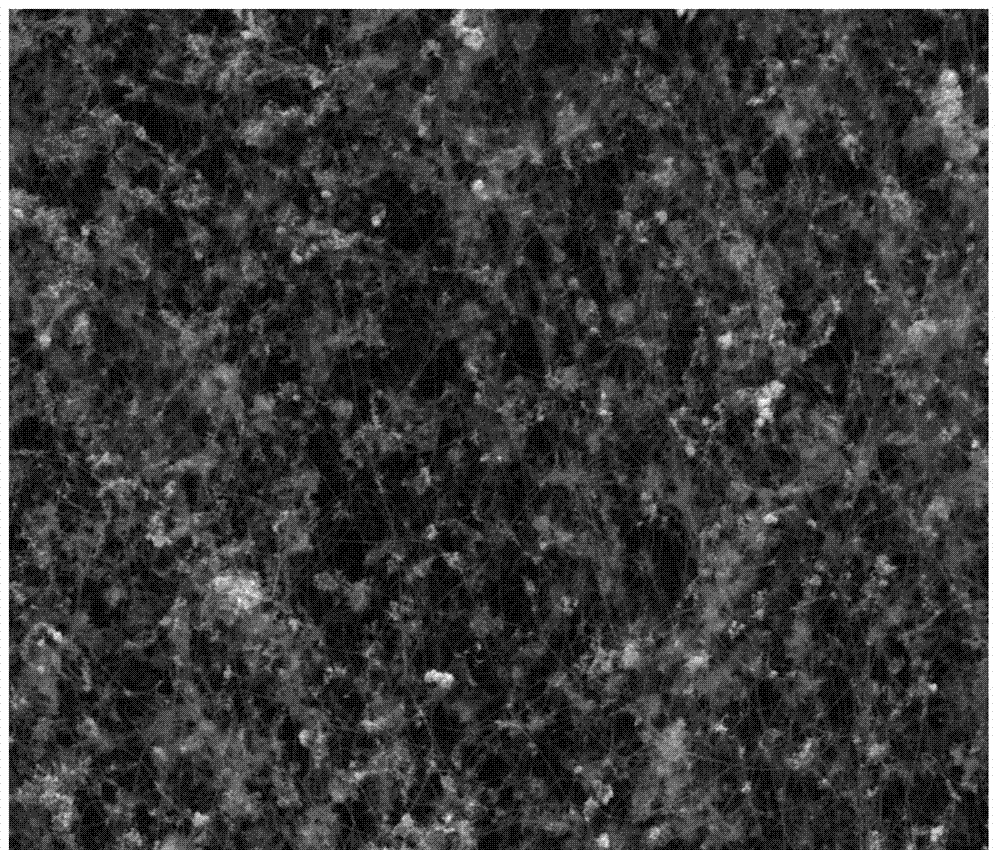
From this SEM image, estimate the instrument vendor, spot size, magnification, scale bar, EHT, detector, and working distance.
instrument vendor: FEI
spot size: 3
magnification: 10 K X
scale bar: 5000 nm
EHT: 10 kV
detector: ETD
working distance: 8 mm